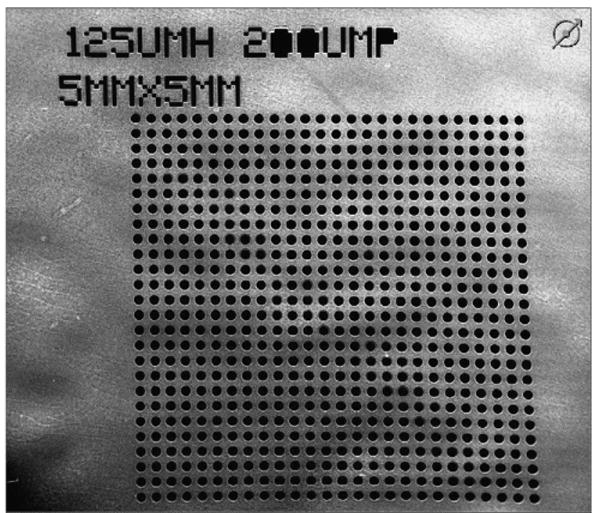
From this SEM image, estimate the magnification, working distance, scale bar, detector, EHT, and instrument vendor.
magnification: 0.037 K X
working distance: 43.2 mm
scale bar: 3000000 nm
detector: ETD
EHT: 30 kV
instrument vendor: FEI